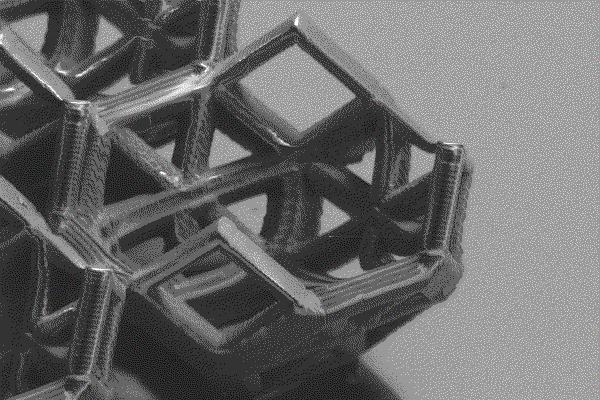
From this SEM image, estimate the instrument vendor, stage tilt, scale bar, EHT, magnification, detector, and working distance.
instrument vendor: Zeiss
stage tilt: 55°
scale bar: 20000 nm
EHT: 3 kV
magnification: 1.89 K X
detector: SE2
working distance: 8.5 mm